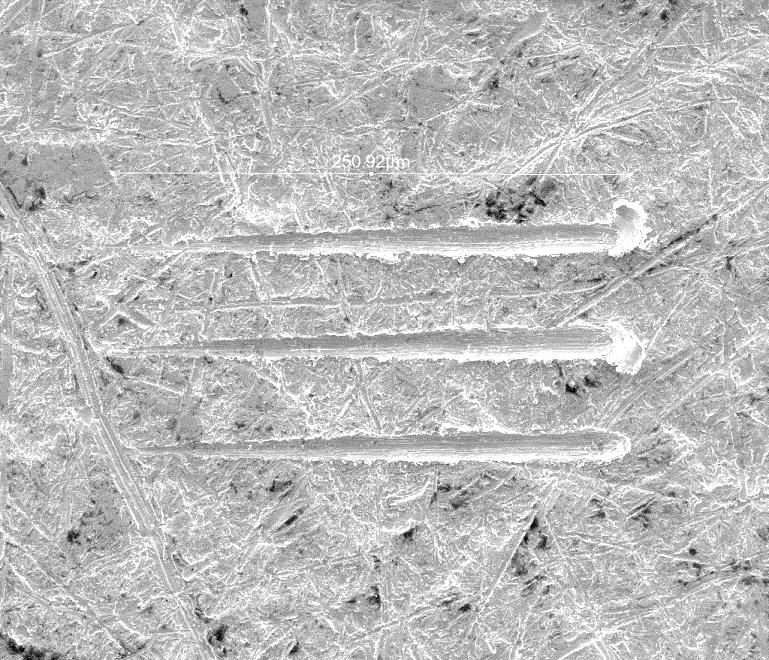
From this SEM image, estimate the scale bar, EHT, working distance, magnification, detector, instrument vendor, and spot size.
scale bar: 200000 nm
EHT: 10 kV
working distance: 10.2 mm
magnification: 0.8 K X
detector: ETD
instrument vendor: FEI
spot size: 3.5